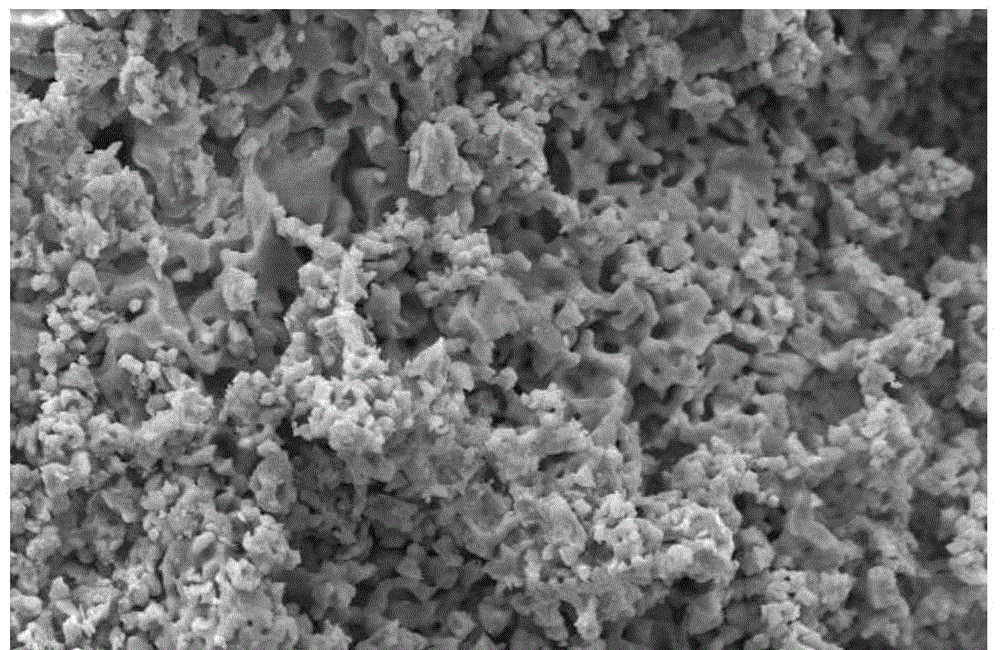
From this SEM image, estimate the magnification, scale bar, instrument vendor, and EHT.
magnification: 1 K X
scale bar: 10000 nm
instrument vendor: JEOL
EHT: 20 kV